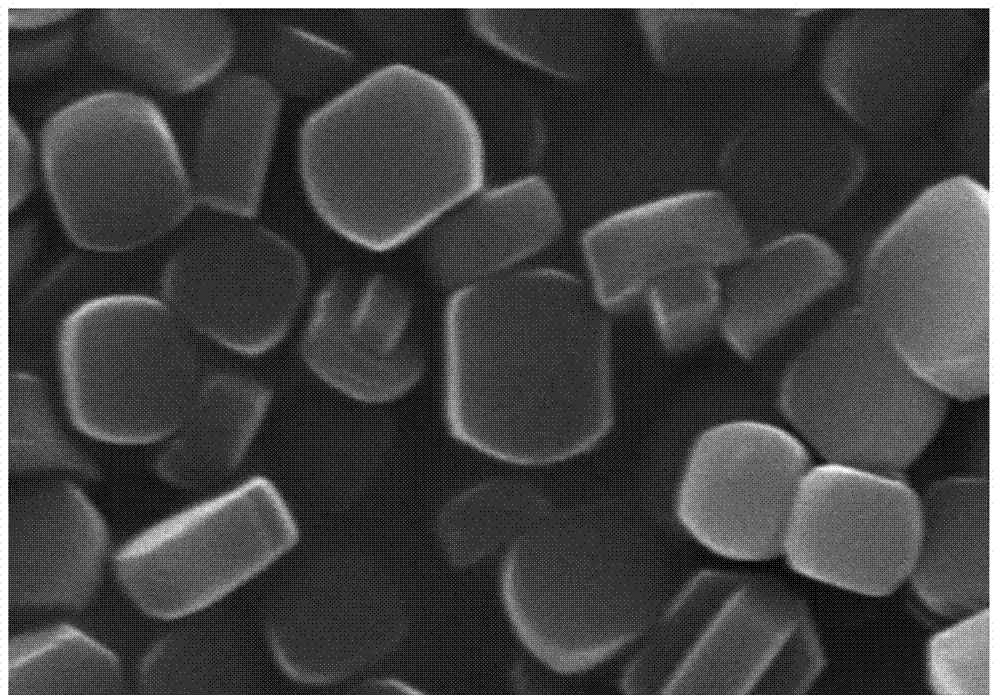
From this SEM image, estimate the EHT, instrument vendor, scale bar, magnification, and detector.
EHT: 15 kV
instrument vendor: Hitachi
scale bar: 2000 nm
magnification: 25 K X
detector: SE